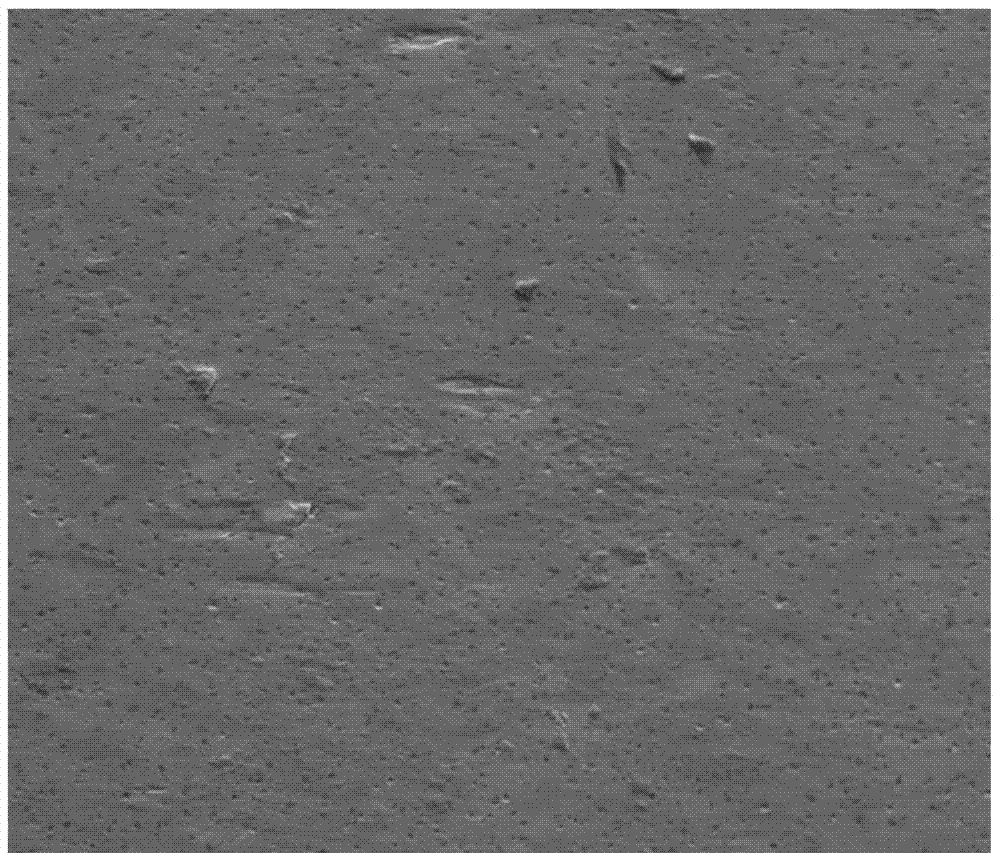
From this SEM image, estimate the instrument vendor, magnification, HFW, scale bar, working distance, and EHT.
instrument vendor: FEI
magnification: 0.5 K X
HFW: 597 µm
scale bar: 200000 nm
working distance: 12.9 mm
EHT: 20 kV